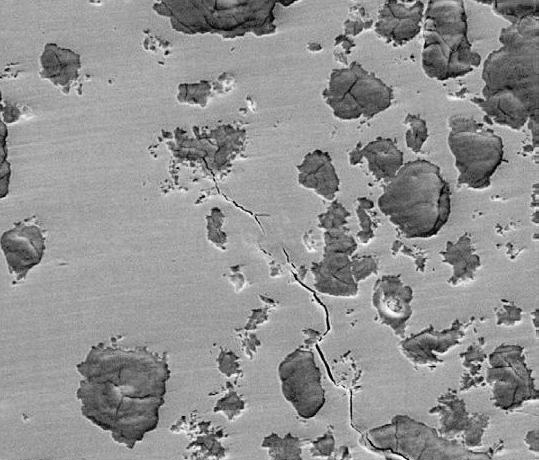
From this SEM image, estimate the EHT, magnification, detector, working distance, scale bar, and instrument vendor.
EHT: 25 kV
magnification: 0.5 K X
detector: ETD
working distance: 10.4 mm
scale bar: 100000 nm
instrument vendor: FEI